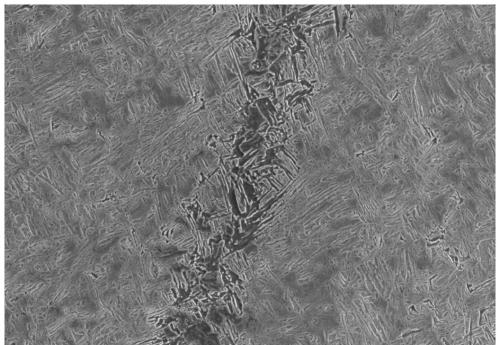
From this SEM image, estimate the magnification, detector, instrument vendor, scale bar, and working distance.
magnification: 1 K X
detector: SE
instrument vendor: Hitachi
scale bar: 50000 nm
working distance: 9.5 mm